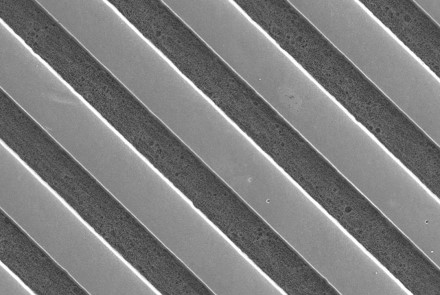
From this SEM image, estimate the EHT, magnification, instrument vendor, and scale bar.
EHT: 30 kV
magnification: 0.5 K X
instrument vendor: JEOL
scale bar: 50000 nm